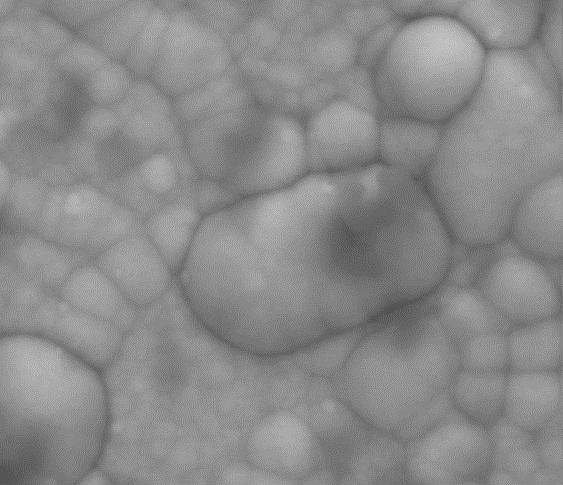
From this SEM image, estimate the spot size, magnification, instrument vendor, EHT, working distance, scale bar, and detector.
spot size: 5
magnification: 3 K X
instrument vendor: FEI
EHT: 30 kV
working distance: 9.7 mm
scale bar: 20000 nm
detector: Mix